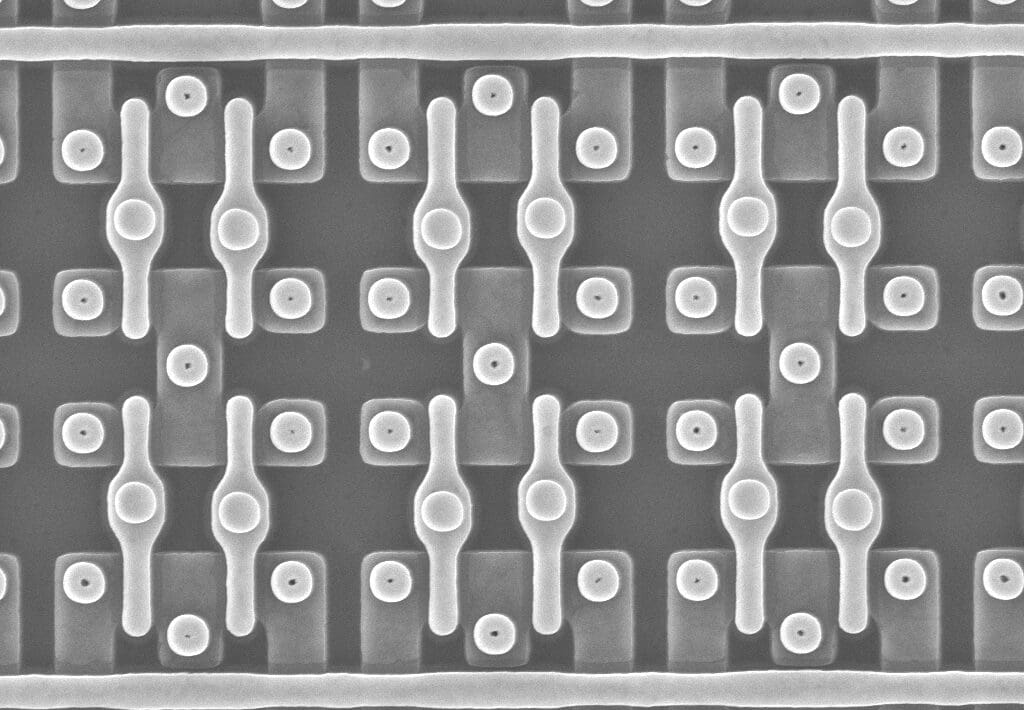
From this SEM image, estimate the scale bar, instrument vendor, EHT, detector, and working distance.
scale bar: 1000 nm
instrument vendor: Zeiss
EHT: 10 kV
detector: InLens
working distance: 9.3 mm